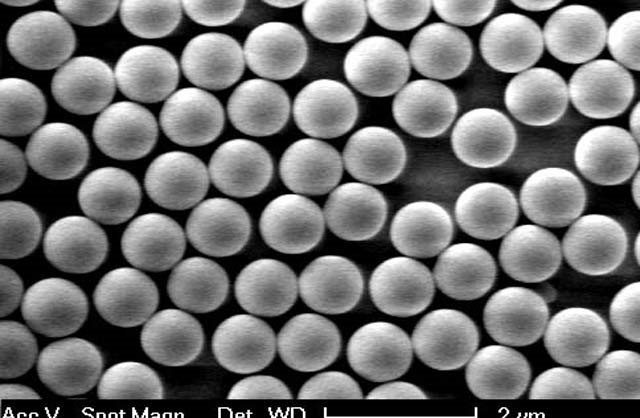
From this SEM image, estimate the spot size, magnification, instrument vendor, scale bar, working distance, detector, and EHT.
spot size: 3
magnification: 10 K X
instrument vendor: FEI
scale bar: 2000 nm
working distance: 5 mm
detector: SE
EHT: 5 kV